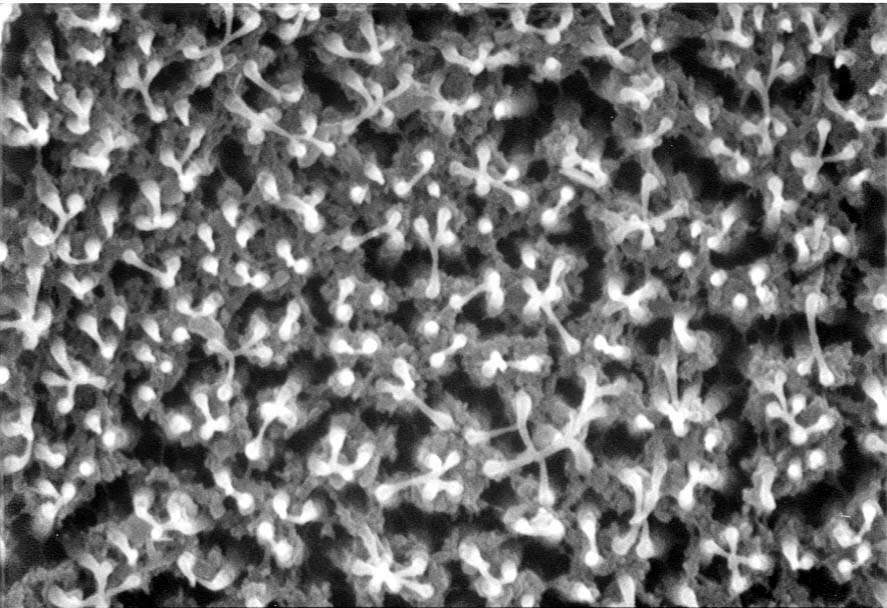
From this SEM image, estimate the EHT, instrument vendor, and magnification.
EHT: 20 kV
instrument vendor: JEOL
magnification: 10 K X